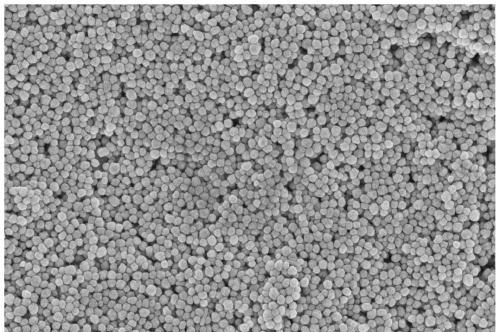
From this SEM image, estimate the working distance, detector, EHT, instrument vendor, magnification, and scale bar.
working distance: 4 mm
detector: InLens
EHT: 5 kV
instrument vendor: Zeiss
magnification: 30 K X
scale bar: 200 nm